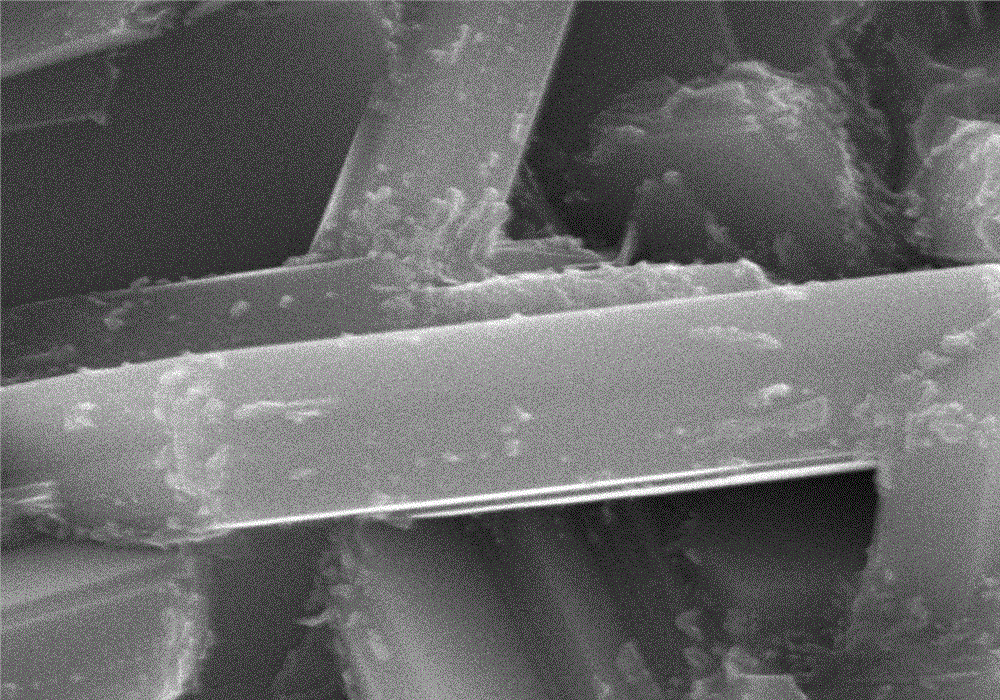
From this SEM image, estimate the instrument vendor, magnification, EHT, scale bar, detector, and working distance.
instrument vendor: Hitachi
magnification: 10 K X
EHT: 30 kV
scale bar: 5000 nm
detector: SE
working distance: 9.1 mm